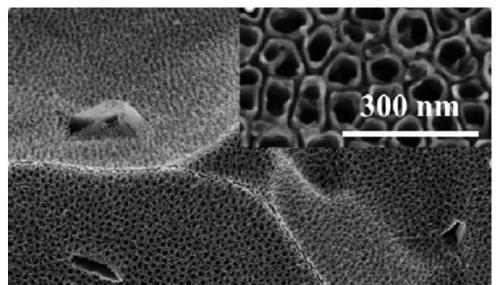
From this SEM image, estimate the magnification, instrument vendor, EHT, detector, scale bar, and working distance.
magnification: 20 K X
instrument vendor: Hitachi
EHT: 5 kV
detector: SE(M)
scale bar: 2000 nm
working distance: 8.4 mm